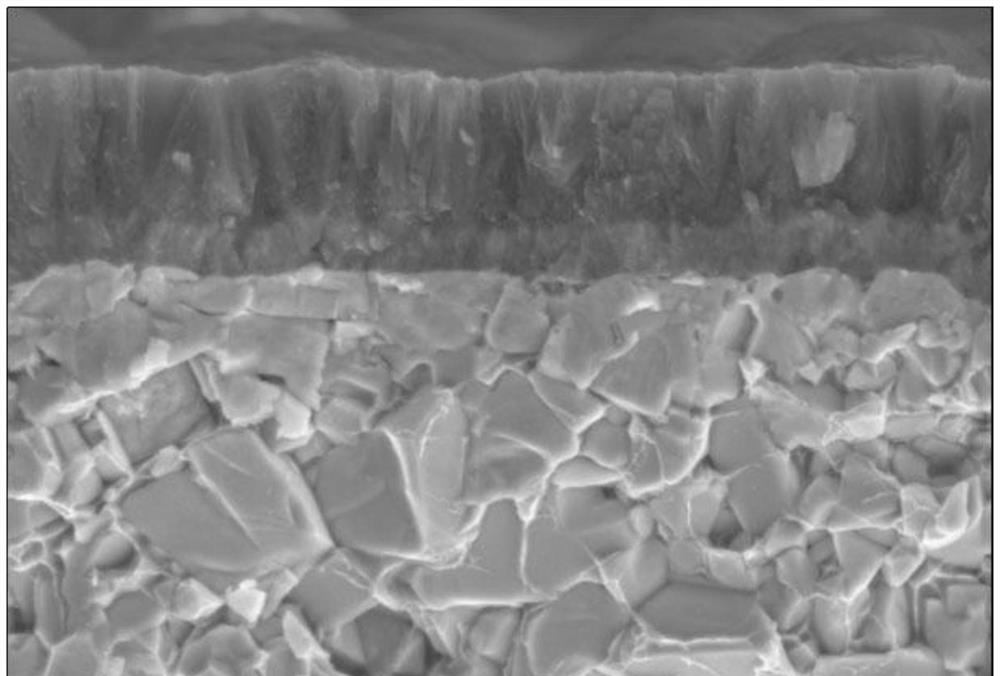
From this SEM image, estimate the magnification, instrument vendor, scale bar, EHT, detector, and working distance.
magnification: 10 K X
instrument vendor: Zeiss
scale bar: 1000 nm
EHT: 10 kV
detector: SE2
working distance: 20.7 mm